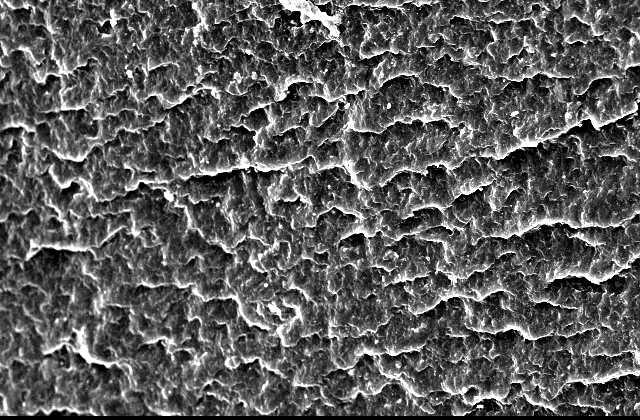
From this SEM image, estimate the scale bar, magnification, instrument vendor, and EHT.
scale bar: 50000 nm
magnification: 0.5 K X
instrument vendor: JEOL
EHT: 15 kV